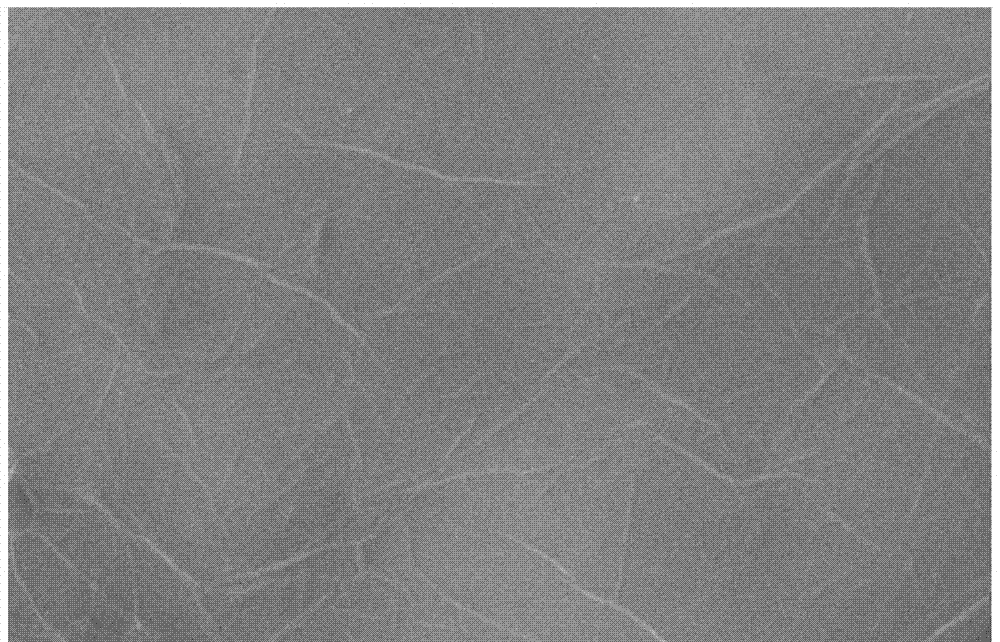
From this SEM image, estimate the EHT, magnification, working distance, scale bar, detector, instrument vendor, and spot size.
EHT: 10 kV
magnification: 10 K X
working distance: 10.1 mm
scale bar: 2000 nm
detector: SE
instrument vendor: FEI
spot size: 5